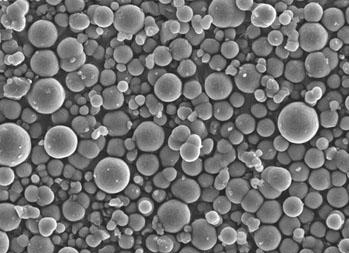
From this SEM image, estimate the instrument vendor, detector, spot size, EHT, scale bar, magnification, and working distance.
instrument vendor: FEI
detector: SE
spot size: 3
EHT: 10 kV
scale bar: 10000 nm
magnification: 2 K X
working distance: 5.2 mm